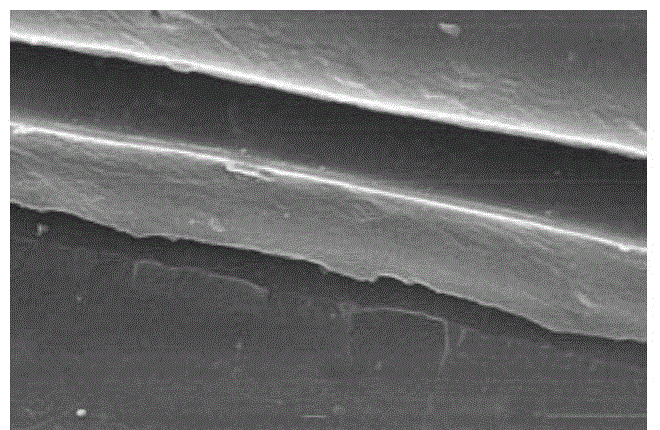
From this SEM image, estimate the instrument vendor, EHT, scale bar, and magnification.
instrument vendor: JEOL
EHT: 15 kV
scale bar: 5000 nm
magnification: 5 K X